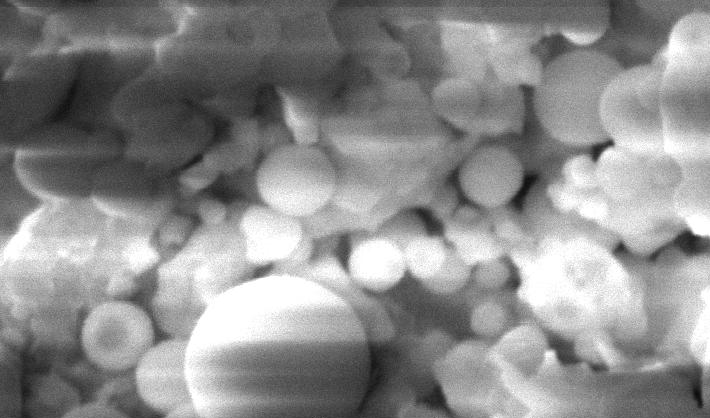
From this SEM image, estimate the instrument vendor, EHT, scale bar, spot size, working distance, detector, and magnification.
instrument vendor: FEI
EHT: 20 kV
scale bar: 2000 nm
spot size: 4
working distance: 11.8 mm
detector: SE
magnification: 10 K X